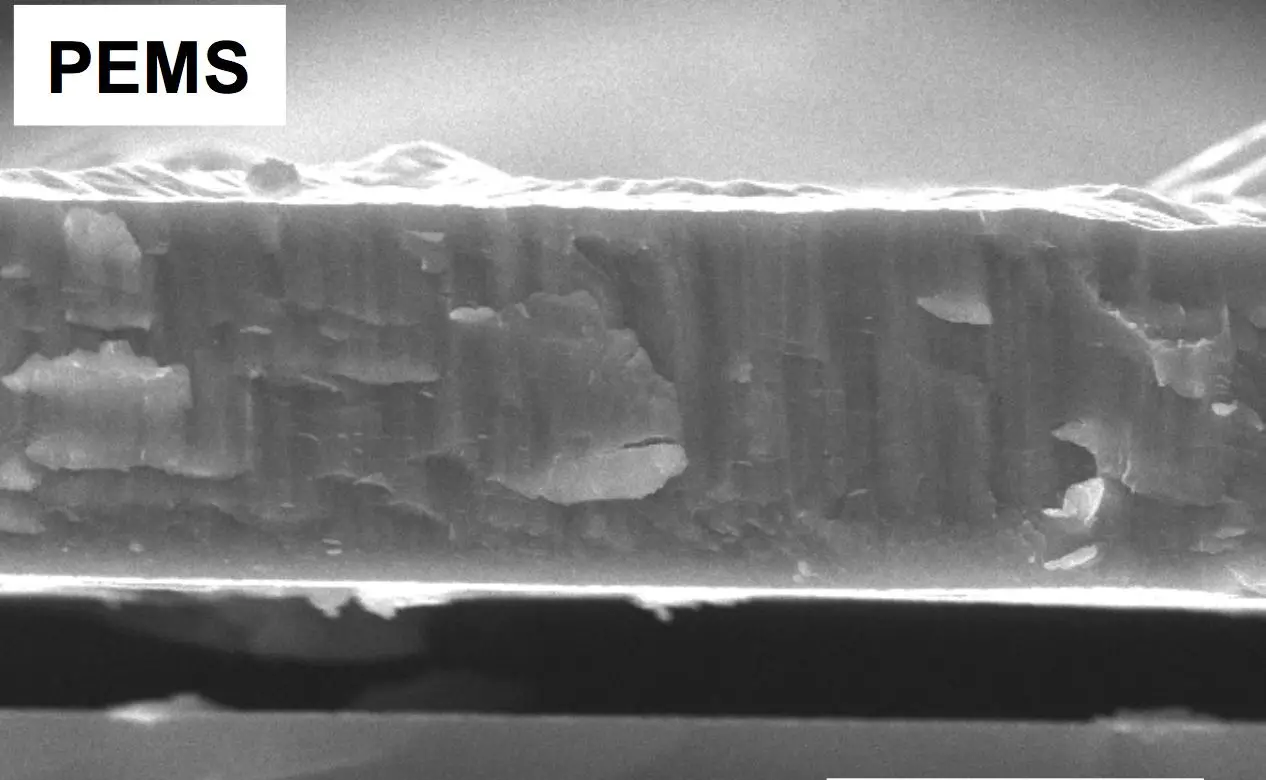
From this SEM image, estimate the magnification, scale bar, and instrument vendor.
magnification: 5 K X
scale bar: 5000 nm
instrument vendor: FEI